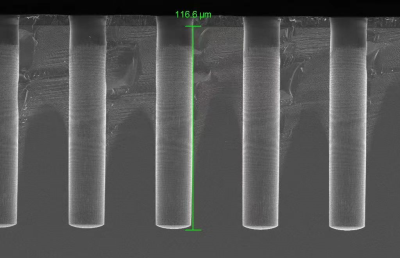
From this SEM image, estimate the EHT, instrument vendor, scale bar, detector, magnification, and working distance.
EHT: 5 kV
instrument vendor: Zeiss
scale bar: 20000 nm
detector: InLens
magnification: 0.5 K X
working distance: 5.7 mm